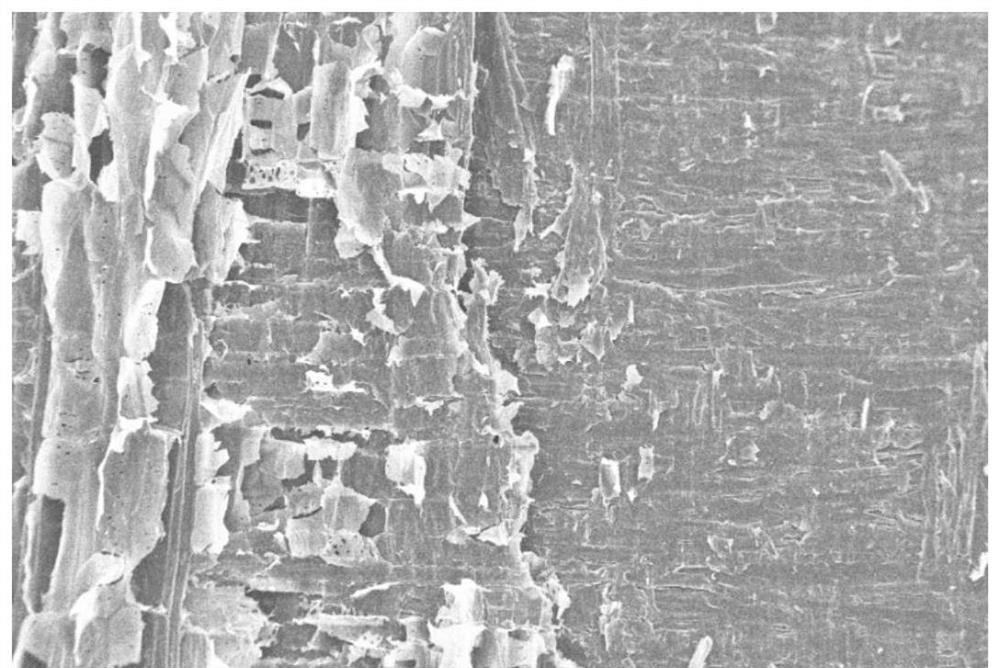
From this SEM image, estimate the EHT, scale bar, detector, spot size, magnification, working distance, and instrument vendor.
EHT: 12 kV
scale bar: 100000 nm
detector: SE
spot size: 9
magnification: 0.2 K X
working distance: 8.6 mm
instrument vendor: other